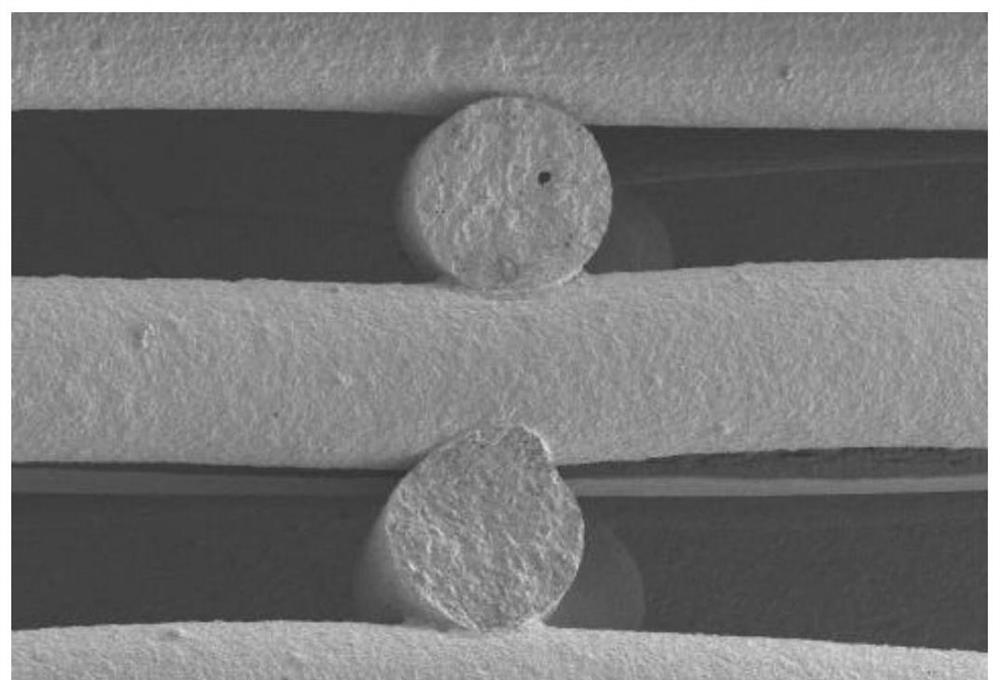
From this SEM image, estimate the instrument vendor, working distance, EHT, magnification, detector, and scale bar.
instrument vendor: Hitachi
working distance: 8.8 mm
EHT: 5 kV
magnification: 0.03 K X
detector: LM(L)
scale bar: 1000000 nm